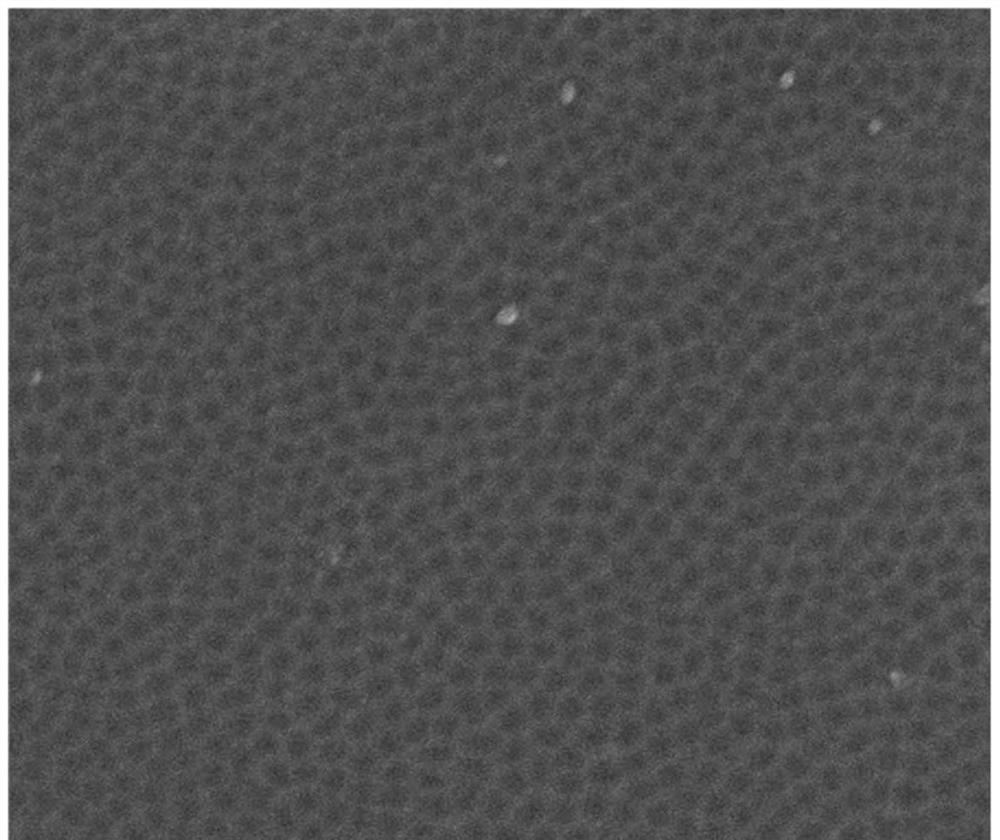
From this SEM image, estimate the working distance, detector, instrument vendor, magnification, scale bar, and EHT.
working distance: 6.2 mm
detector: ETD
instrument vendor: FEI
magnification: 30 K X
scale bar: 1000 nm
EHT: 15 kV